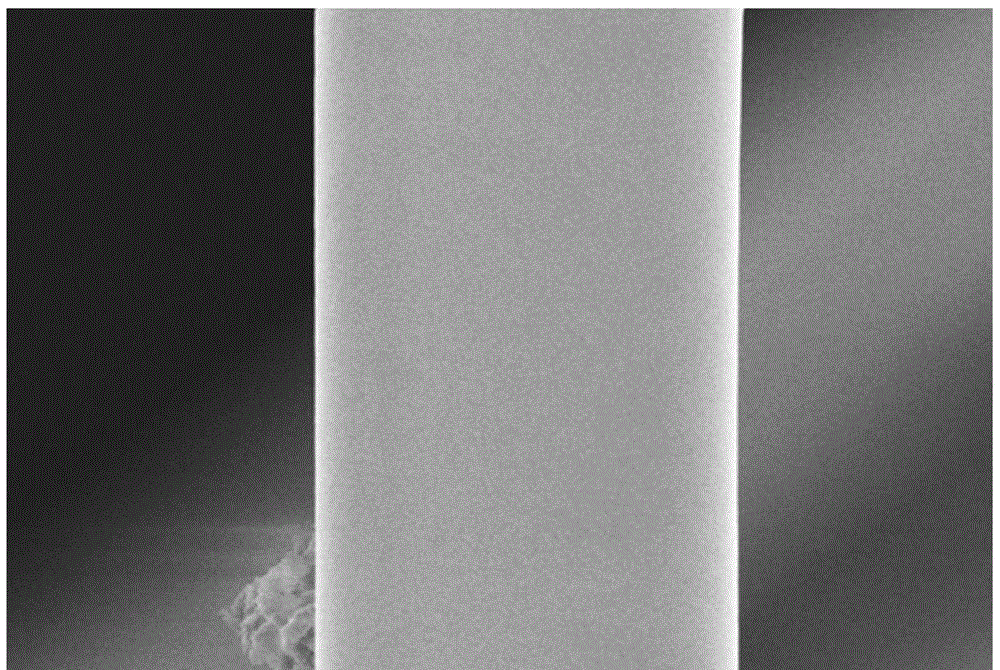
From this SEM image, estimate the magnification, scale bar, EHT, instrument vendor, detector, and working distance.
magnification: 20 K X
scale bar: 2000 nm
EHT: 5 kV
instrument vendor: Zeiss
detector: InLens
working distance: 7.6 mm